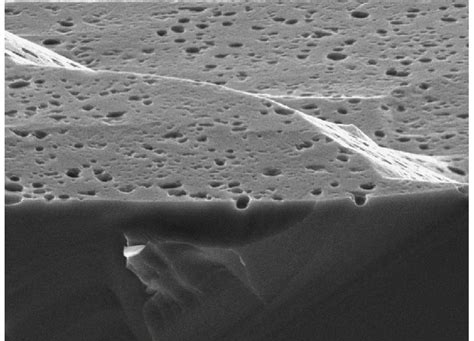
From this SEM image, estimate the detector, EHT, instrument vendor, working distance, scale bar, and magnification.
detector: SEI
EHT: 5 kV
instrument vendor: JEOL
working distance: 10 mm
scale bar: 1000 nm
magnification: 15 K X